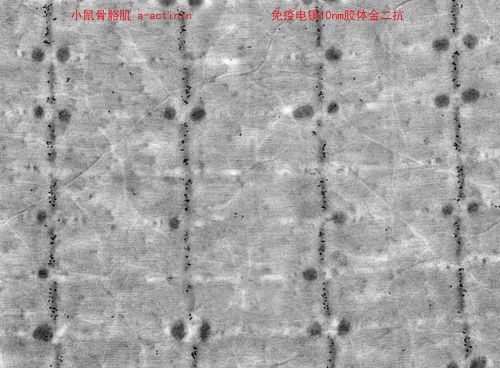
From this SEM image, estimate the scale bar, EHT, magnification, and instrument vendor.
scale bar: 2000 nm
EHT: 80 kV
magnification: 5 K X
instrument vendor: Hitachi